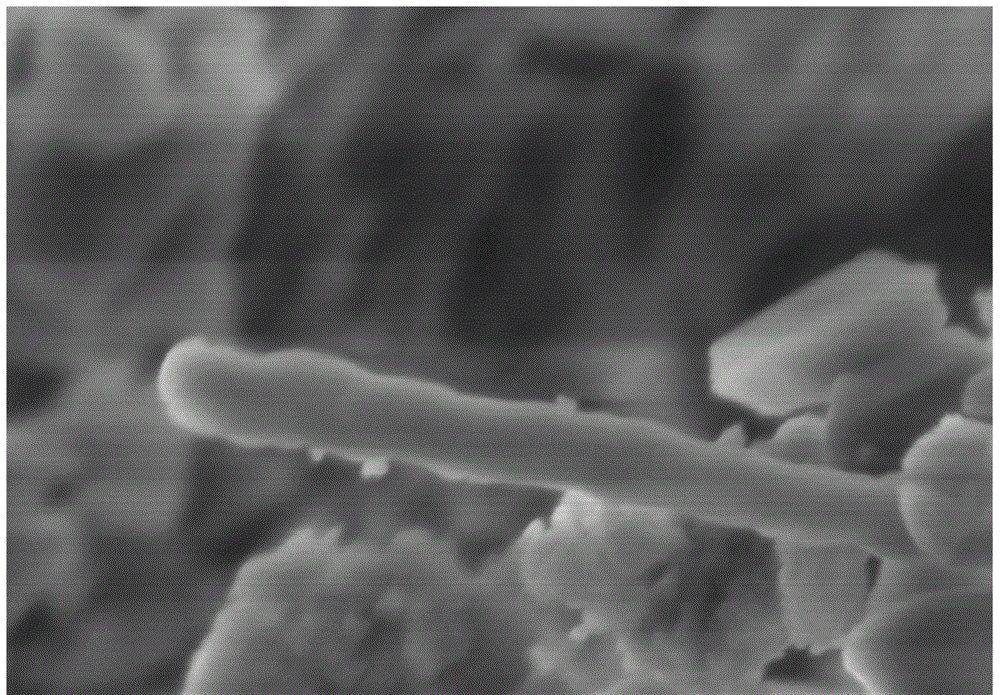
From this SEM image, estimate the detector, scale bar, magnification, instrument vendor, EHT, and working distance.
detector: SE(M)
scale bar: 500 nm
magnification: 100 K X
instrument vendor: Hitachi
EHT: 3 kV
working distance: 5.6 mm